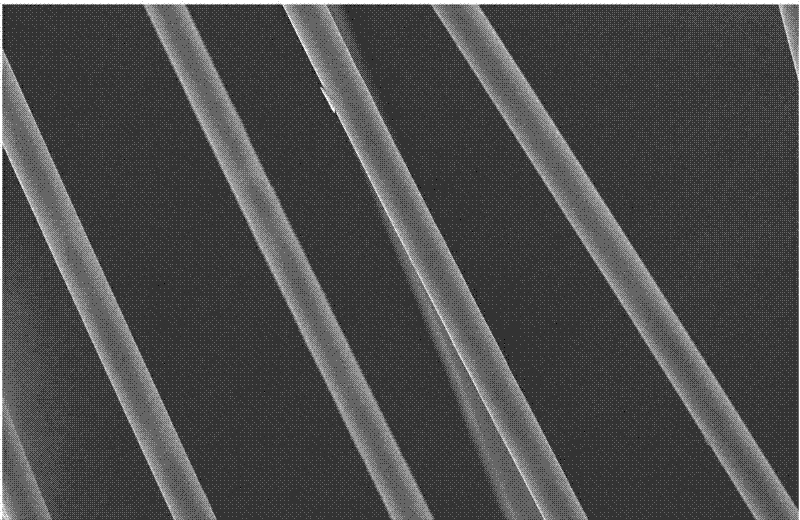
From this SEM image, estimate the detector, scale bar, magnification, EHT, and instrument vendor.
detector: SEI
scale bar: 50000 nm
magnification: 0.5 K X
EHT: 25 kV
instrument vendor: JEOL